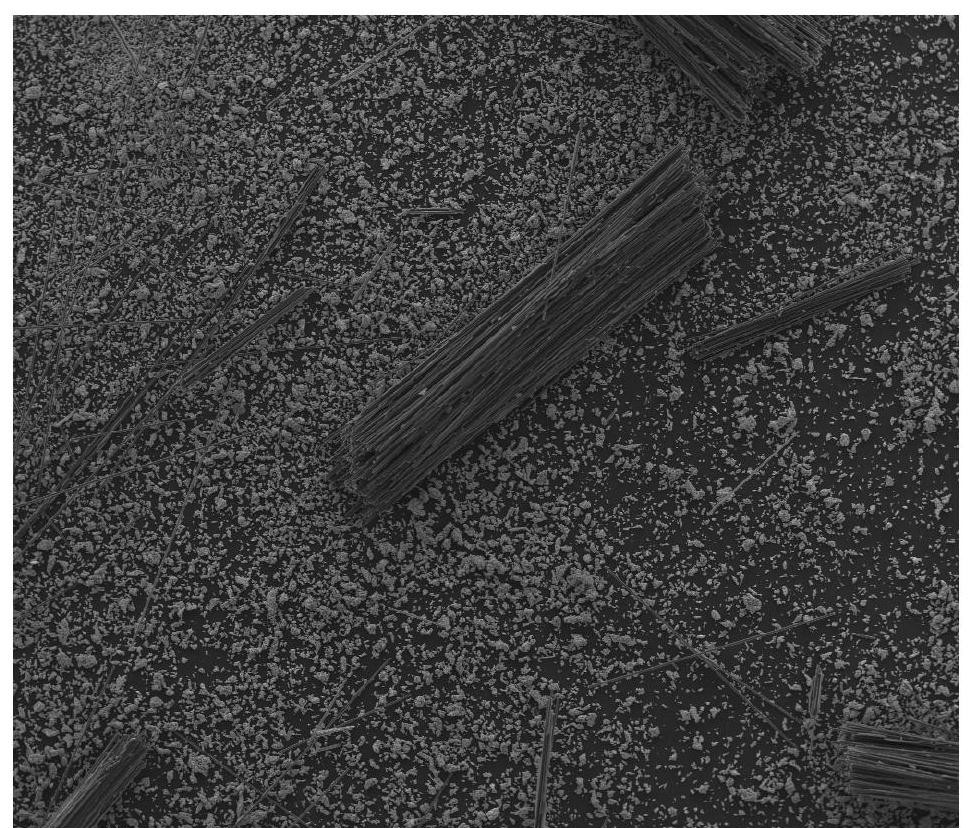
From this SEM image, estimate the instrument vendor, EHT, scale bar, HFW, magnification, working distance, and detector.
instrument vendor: FEI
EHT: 20 kV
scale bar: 1000000 nm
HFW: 2980 µm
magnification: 0.1 K X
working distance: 10.9 mm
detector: ETD